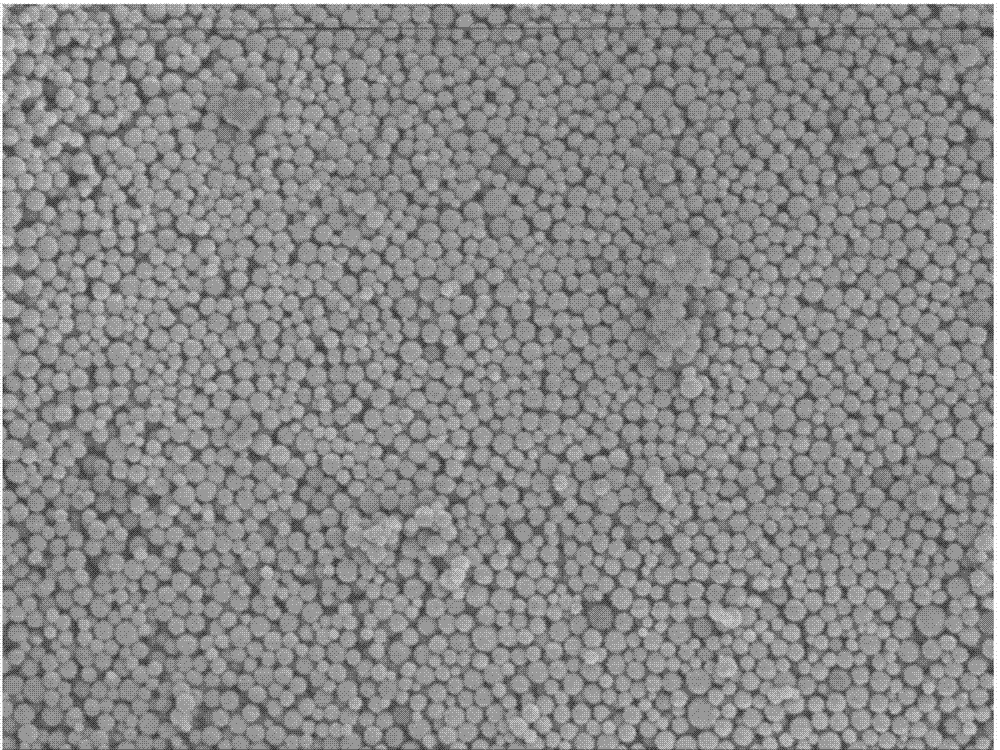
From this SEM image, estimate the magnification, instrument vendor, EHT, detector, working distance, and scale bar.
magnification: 10 K X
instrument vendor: JEOL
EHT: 3 kV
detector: SEI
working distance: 37.2 mm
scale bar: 1000 nm